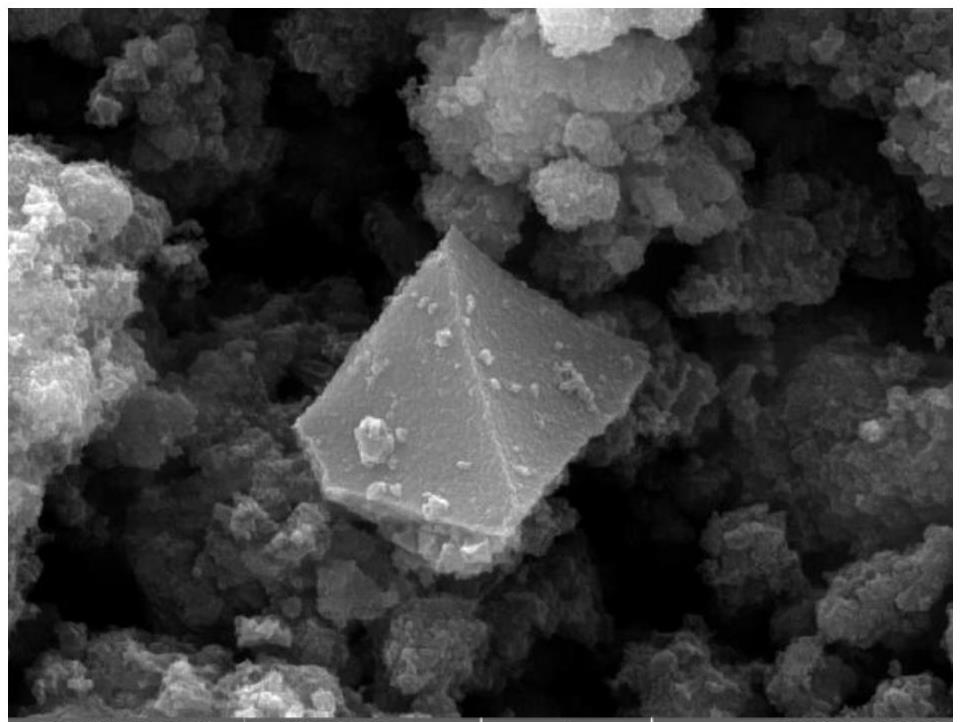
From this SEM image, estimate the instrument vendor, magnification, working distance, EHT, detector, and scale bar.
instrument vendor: Tescan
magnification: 50 K X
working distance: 16.22 mm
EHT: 20 kV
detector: SE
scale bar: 1000 nm